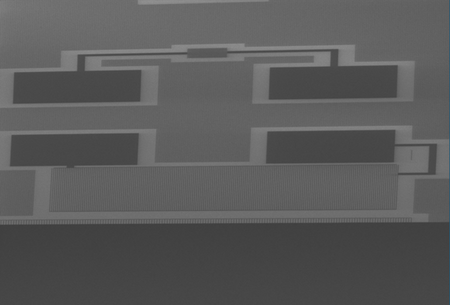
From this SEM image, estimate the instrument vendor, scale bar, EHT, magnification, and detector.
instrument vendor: Hitachi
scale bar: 100000 nm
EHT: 5 kV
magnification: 0.45 K X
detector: SE